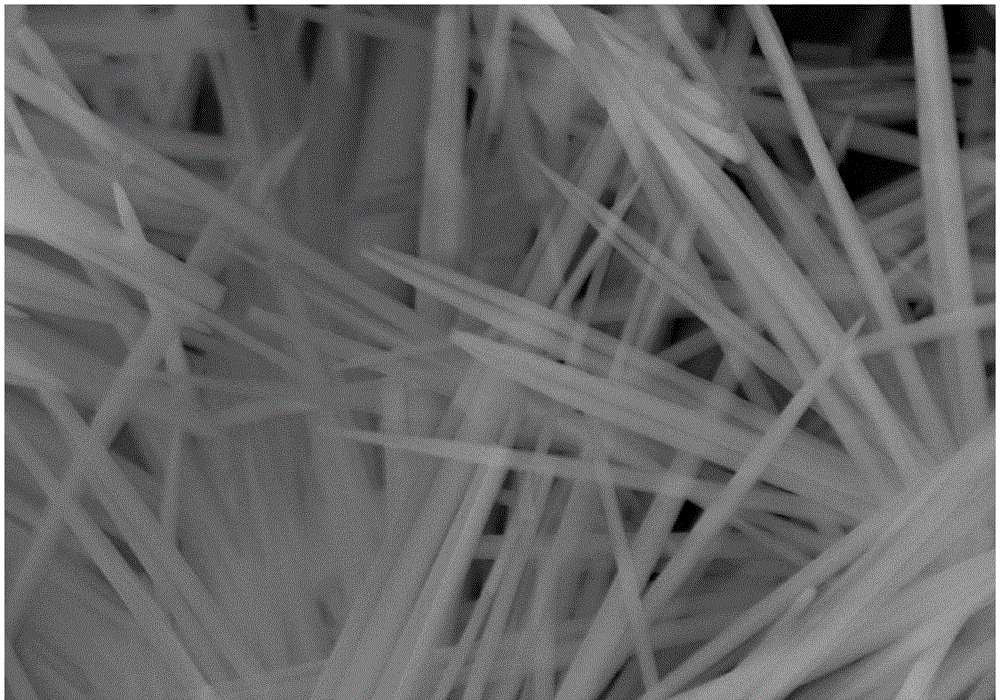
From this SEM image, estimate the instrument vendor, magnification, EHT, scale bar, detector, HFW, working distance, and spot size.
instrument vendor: FEI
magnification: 80 K X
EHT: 20 kV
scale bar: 2000 nm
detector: ETD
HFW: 5.18 µm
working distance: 9.4 mm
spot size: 3